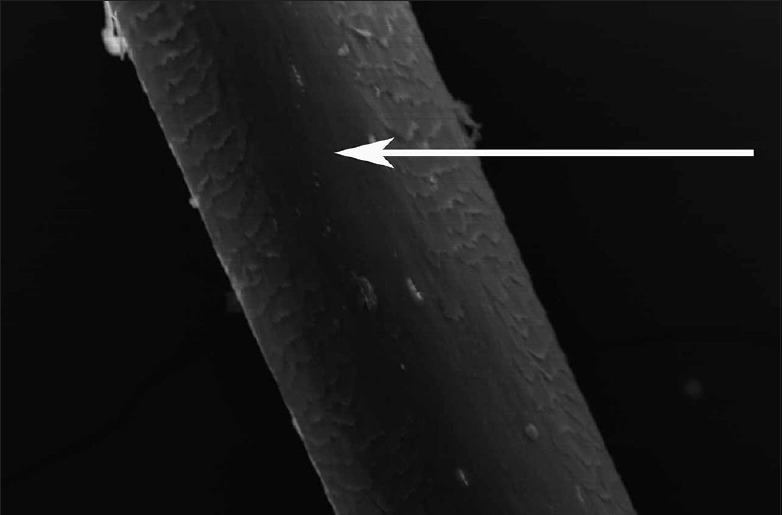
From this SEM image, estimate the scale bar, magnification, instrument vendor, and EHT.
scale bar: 50000 nm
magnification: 0.5 K X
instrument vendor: JEOL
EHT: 15 kV